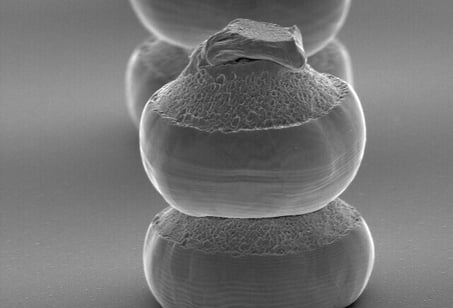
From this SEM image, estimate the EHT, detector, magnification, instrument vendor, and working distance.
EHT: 20 kV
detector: SE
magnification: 0.75 K X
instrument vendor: Hitachi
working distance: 22.5 mm